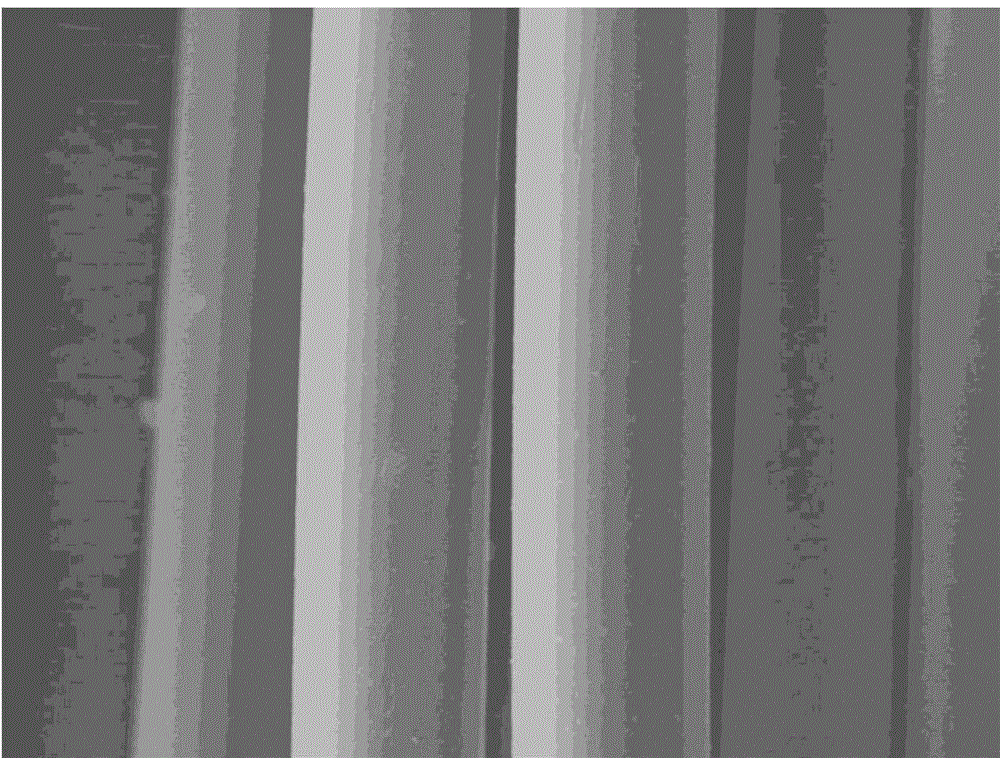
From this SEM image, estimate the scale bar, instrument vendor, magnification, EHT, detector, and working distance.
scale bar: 10000 nm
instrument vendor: JEOL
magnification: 1 K X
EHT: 20 kV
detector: LEI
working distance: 14.9 mm